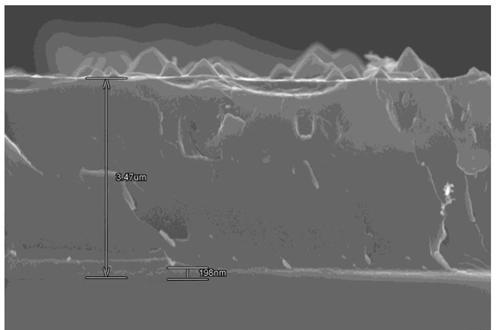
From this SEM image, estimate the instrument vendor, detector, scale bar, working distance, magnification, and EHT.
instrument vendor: Hitachi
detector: SE(U)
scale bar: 3000 nm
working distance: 11 mm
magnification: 15 K X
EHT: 15 kV